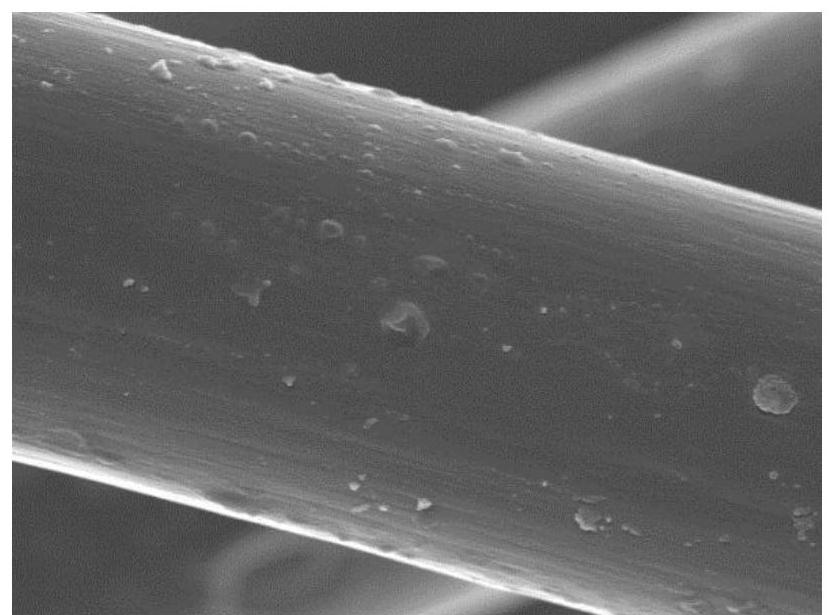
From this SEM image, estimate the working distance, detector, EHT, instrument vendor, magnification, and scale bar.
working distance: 7.1 mm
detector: SEI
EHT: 5 kV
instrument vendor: JEOL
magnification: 10 K X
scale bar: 1000 nm